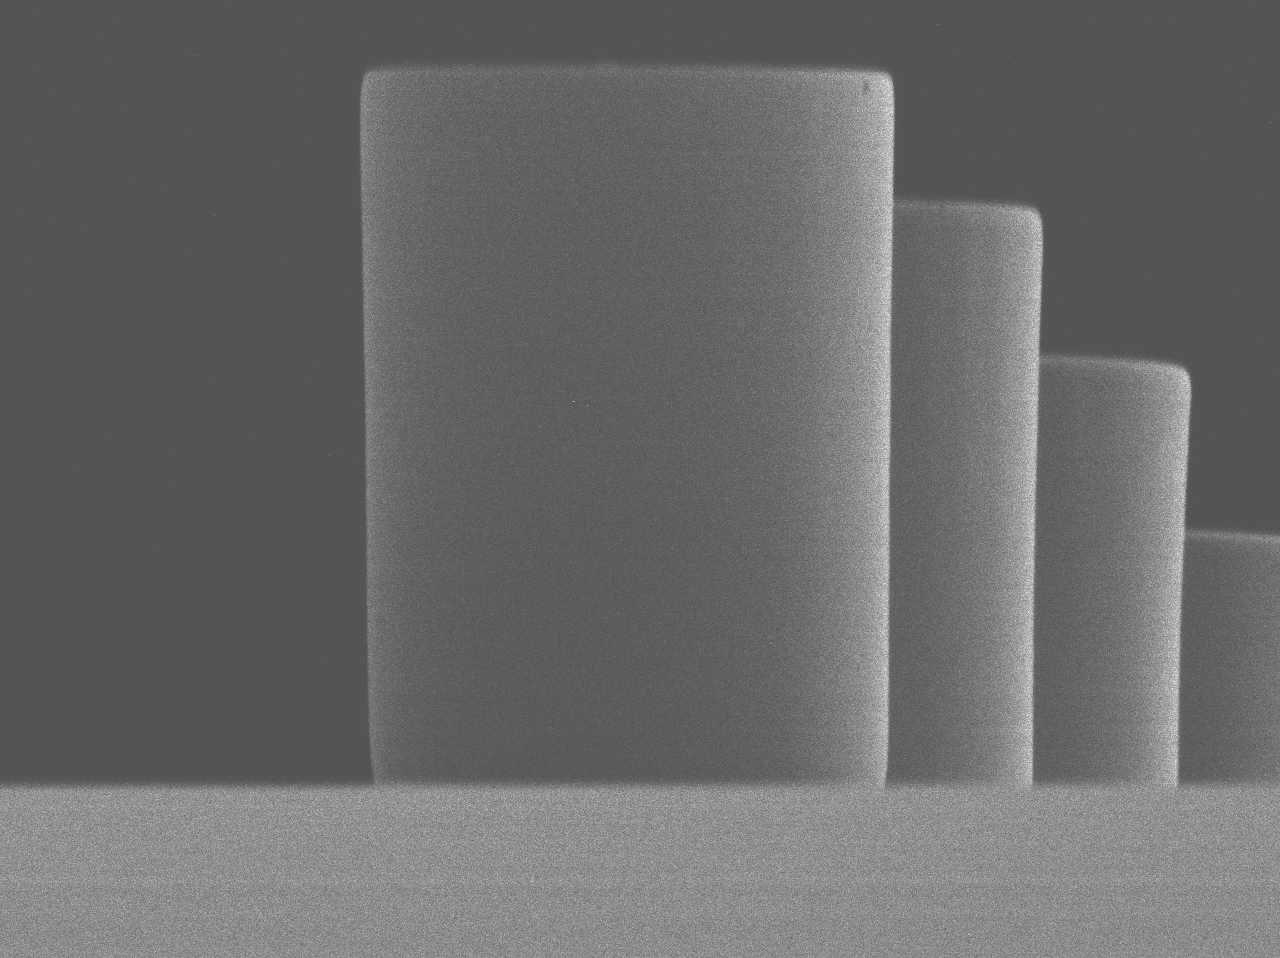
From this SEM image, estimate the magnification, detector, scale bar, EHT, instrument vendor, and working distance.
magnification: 0.95 K X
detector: LEI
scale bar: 10000 nm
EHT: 2 kV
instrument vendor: JEOL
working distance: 8 mm